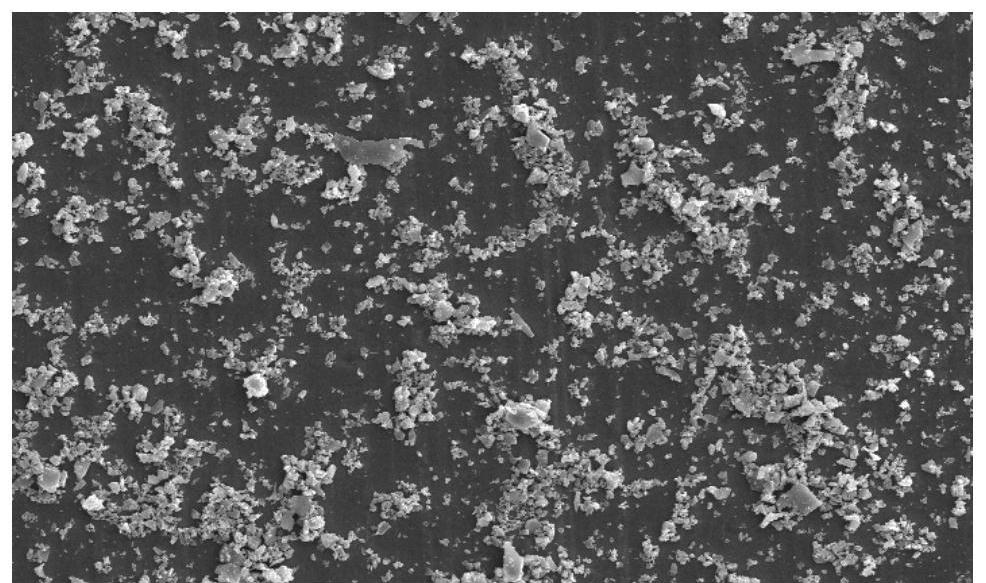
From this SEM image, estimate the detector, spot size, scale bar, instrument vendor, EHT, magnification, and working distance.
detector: SE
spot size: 4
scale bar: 50000 nm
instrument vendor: FEI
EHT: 25 kV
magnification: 1 K X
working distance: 7.7 mm